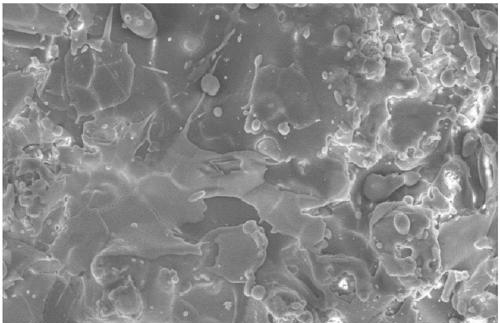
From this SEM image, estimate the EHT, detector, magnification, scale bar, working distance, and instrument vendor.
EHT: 15 kV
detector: SE(M)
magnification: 1 K X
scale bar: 50000 nm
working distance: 14.8 mm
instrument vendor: Hitachi